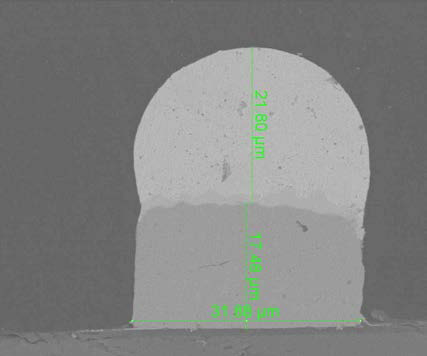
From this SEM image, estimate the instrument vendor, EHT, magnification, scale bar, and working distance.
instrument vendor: FEI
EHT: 10 kV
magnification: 5 K X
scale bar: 30000 nm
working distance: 16.3 mm